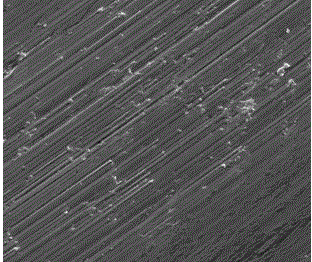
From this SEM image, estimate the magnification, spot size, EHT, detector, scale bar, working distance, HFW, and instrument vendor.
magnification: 1 K X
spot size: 3.5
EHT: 30 kV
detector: ETD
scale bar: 50000 nm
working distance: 13.2 mm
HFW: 298 µm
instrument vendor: FEI